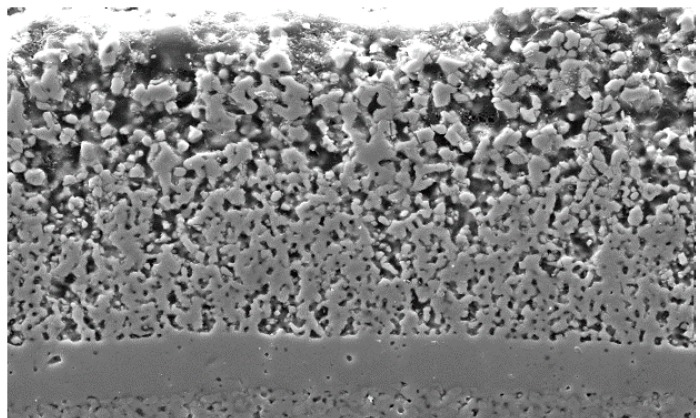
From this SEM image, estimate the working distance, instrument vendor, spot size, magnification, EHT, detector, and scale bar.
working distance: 10 mm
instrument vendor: FEI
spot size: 3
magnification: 2 K X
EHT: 15 kV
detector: SE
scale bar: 10000 nm